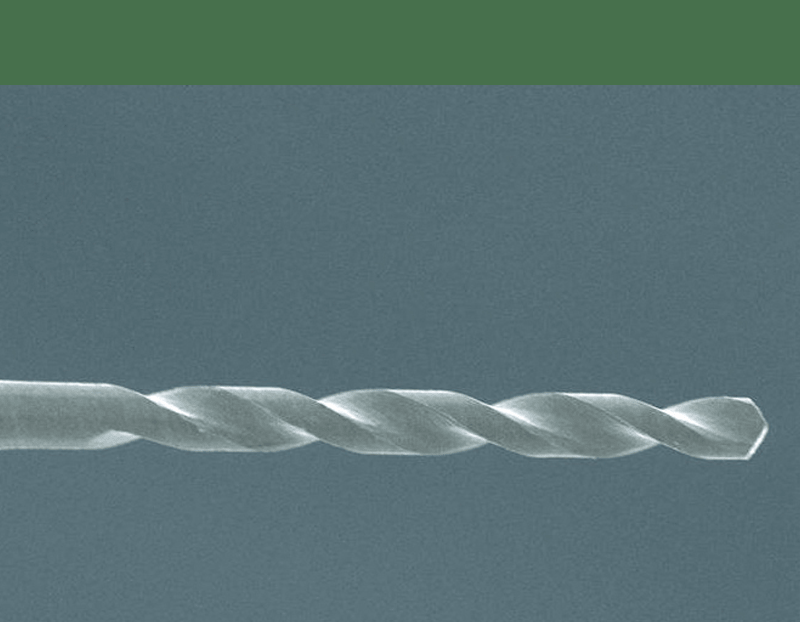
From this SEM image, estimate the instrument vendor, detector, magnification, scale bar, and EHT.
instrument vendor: JEOL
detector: SEI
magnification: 1 K X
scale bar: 10000 nm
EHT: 10 kV